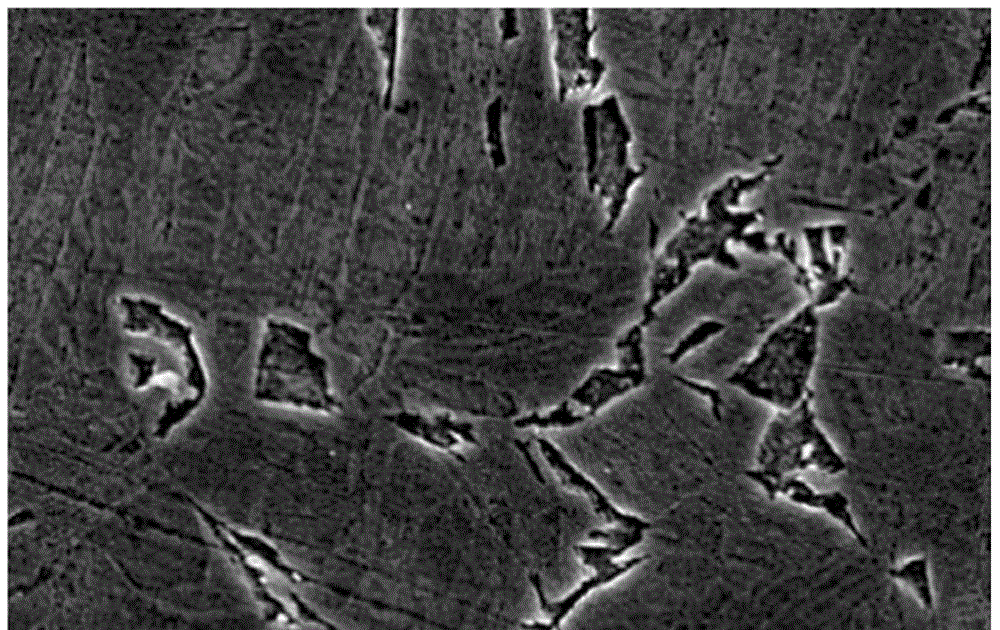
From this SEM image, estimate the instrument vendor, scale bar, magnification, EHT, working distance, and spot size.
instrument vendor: other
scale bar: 1000 nm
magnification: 15 K X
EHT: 2 kV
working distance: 7.9 mm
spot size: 3